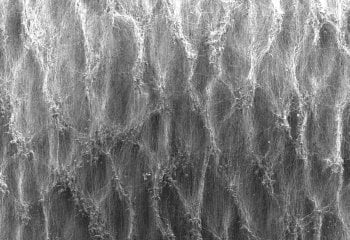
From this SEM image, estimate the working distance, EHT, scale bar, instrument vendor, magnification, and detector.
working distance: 13.2 mm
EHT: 10 kV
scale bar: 1000000 nm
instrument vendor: Hitachi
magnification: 0.04 K X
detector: SE(U)